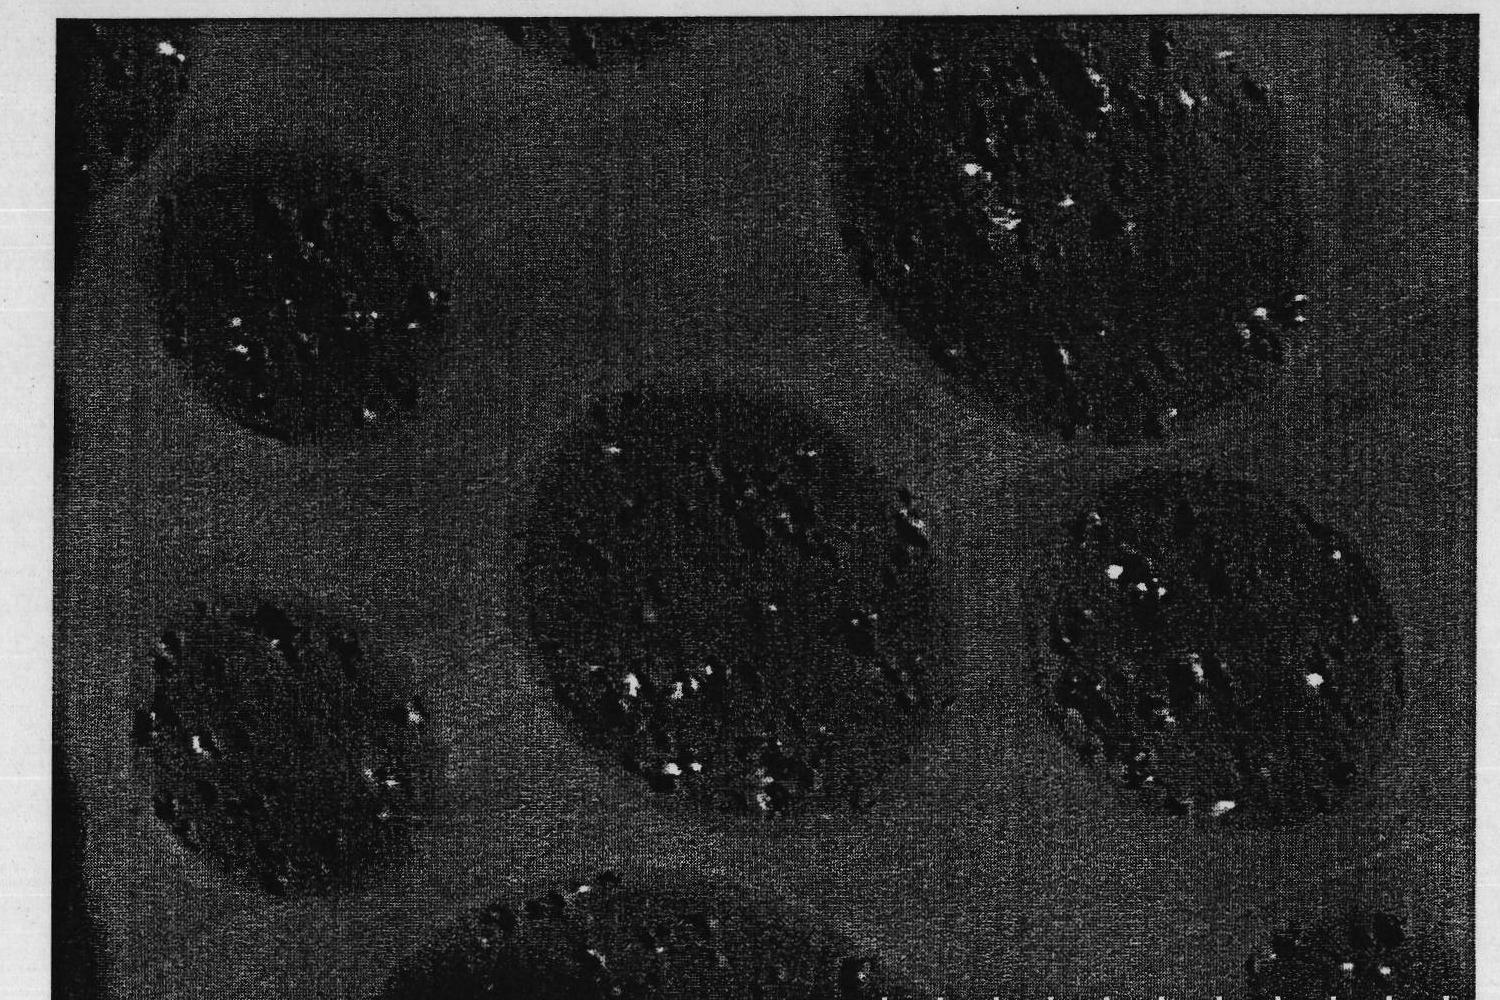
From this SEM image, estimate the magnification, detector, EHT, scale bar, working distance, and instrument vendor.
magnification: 10 K X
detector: TE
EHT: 30 kV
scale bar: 5000 nm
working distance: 8.9 mm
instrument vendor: Hitachi